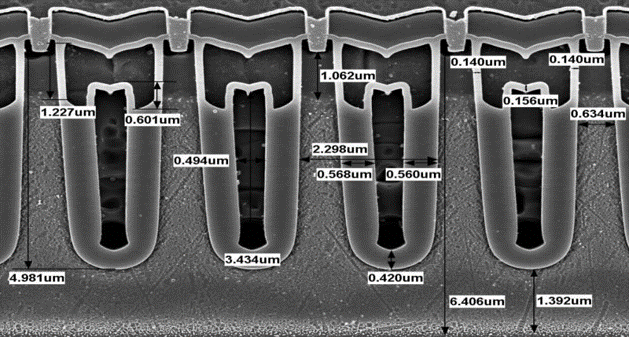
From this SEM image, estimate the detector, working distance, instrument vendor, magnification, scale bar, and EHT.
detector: SE(U)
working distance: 8 mm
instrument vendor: Hitachi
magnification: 12 K X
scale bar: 4000 nm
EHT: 5 kV